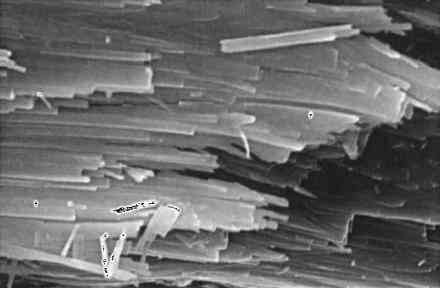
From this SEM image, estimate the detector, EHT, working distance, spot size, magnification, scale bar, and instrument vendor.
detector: SE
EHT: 20 kV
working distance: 10.5 mm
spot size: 3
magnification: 8 K X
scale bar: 5000 nm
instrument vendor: FEI